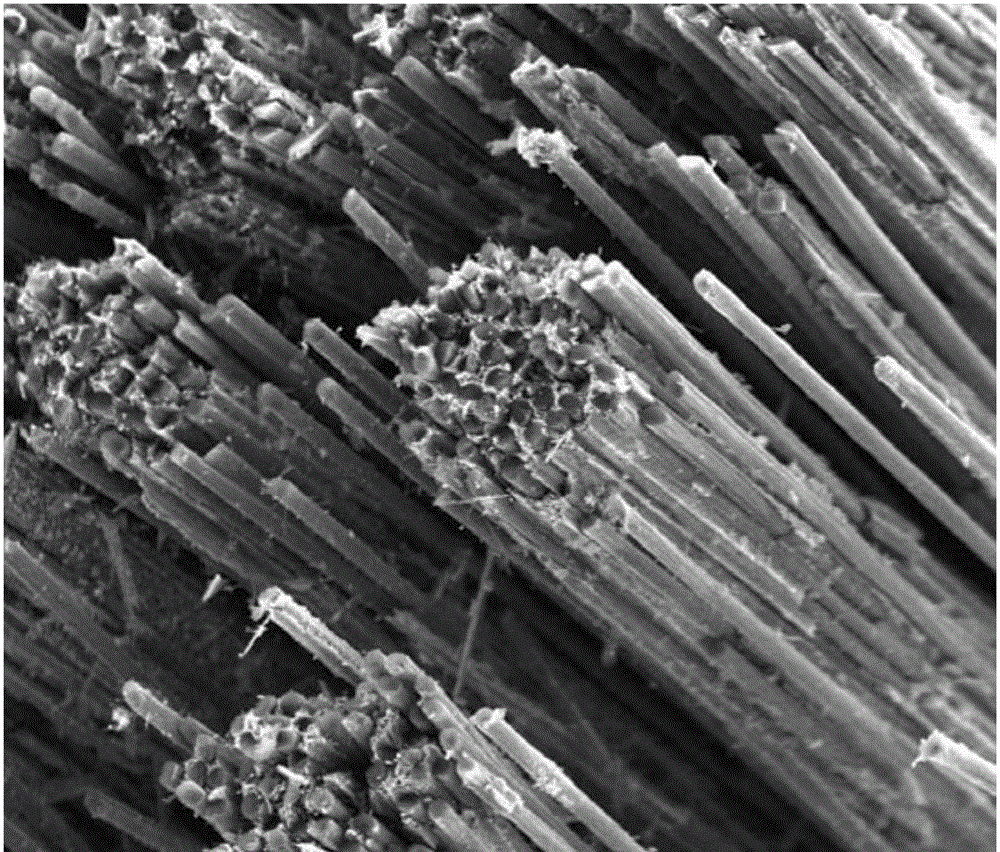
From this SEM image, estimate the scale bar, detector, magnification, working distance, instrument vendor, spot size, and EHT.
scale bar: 100000 nm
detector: ETD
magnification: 0.5 K X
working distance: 8.5 mm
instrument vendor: FEI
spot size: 3.5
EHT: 20 kV